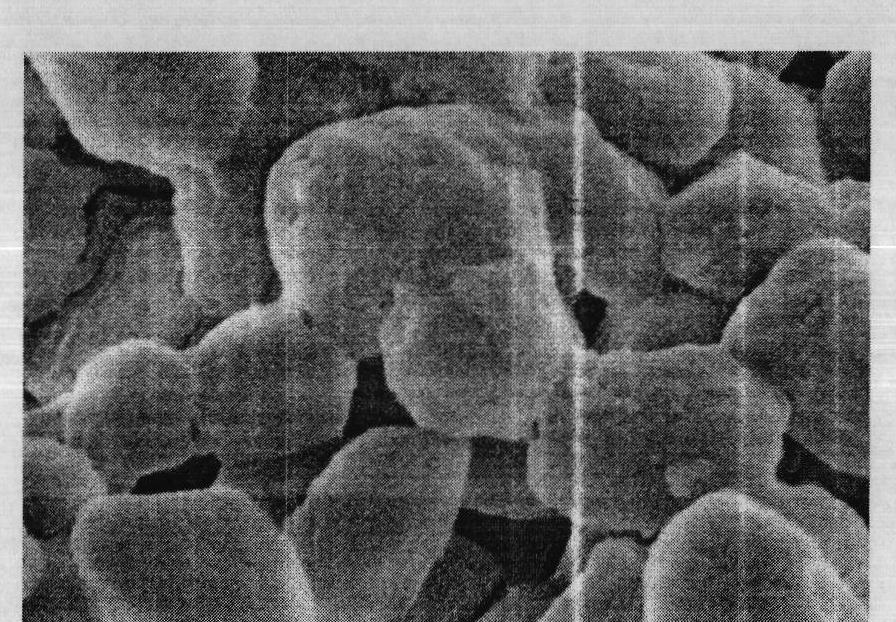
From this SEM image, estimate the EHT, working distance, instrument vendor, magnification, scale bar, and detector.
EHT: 3 kV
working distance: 7.2 mm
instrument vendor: Hitachi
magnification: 80 K X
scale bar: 500 nm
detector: SE(M)